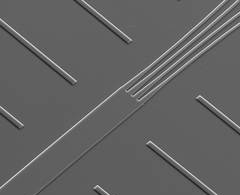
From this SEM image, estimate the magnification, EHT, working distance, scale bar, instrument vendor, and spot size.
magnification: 0.4 K X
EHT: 20 kV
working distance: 11.1 mm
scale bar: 200000 nm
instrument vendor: FEI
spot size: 3